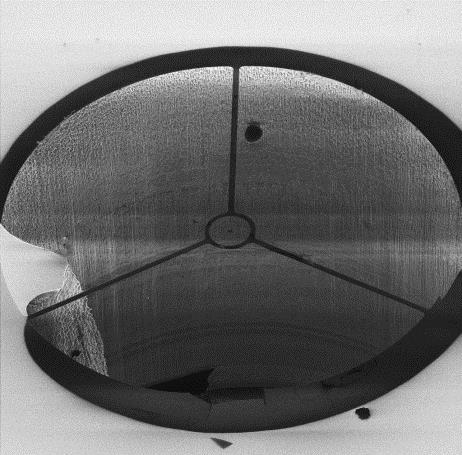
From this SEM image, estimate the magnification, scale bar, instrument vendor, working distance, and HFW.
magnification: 0.66472 K X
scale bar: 20000 nm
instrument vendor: Zeiss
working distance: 8.2 mm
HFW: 300 µm